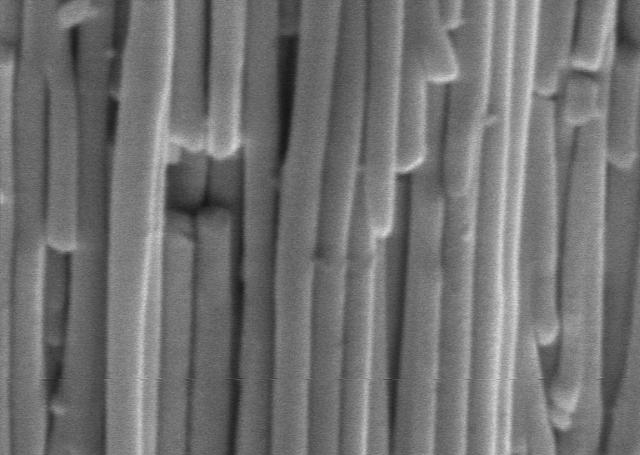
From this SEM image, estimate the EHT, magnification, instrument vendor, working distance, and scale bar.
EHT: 10 kV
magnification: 20 K X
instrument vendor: other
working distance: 6 mm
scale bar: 2000 nm